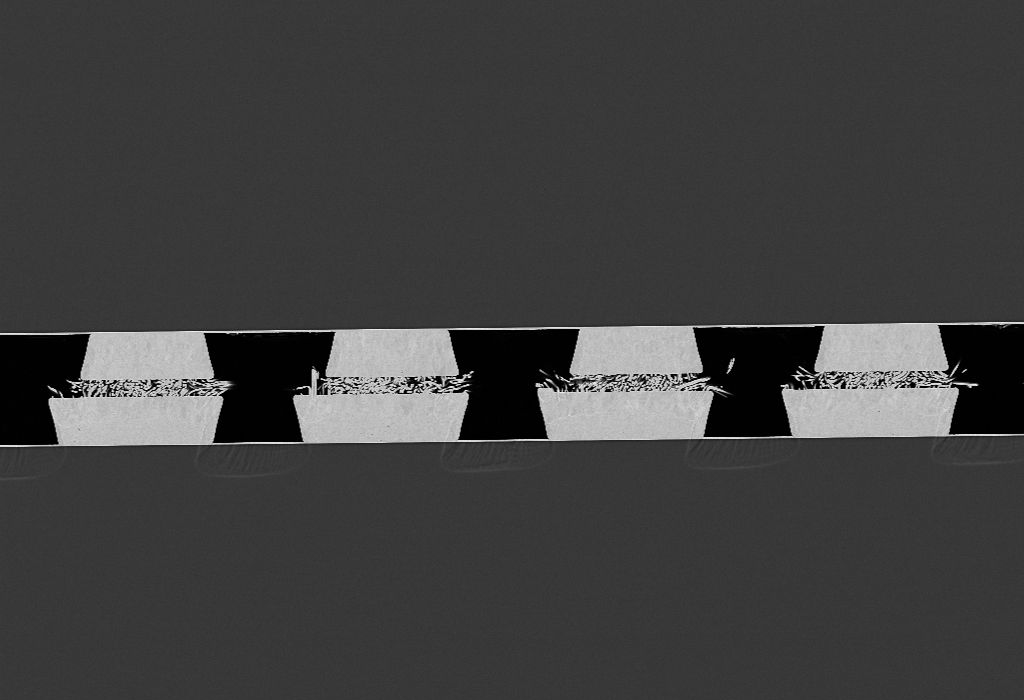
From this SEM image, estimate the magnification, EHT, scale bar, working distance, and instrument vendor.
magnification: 0.5 K X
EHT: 10 kV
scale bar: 20000 nm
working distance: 8 mm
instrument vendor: Zeiss